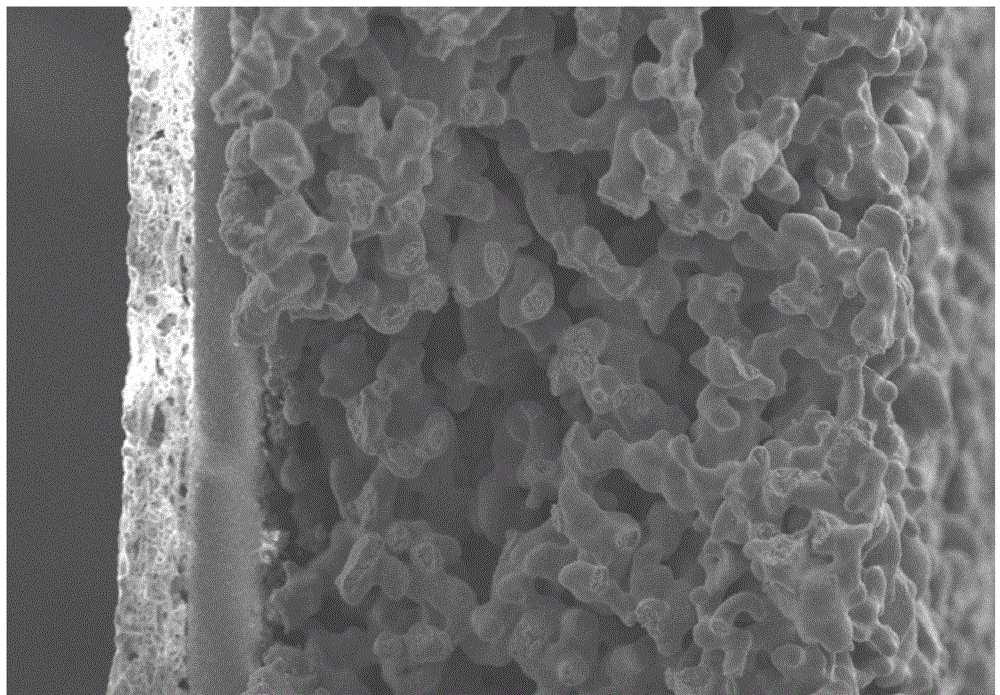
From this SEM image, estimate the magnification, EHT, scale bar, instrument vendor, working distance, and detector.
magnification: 0.3 K X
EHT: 15 kV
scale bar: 100000 nm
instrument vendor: Hitachi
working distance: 4 mm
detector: SE